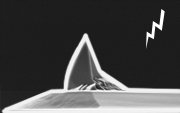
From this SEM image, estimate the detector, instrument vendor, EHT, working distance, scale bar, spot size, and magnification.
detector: SE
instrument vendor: FEI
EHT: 10 kV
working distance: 22.3 mm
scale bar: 10000 nm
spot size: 3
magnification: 3 K X